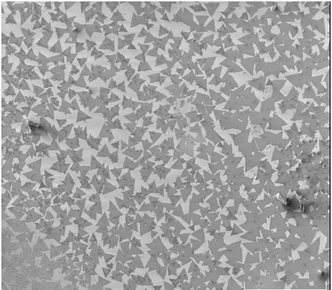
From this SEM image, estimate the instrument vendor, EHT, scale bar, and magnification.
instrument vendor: other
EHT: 1 kV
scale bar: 500000 nm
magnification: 0.05 K X